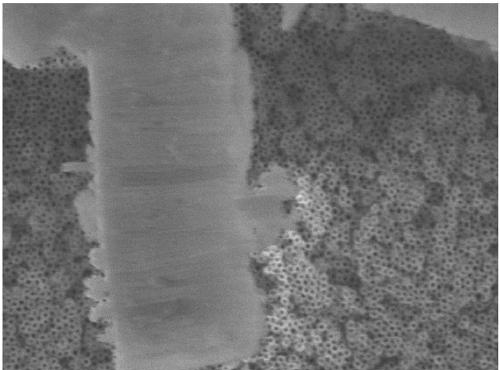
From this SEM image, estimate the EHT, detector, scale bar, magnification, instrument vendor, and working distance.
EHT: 5 kV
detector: SEI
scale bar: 100 nm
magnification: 55 K X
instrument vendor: JEOL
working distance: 6.6 mm